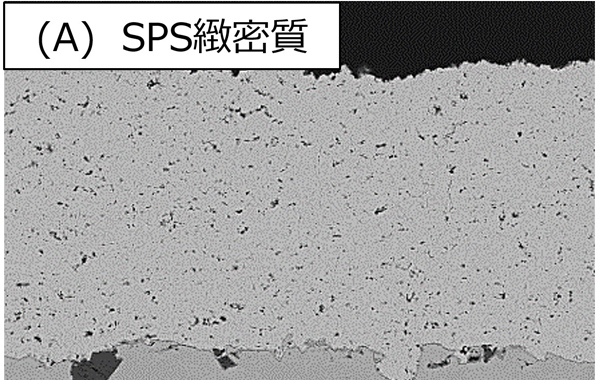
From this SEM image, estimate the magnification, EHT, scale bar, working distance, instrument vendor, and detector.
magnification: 1 K X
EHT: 10 kV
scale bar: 20000 nm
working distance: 15.5 mm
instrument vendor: Hitachi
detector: YAGBSE